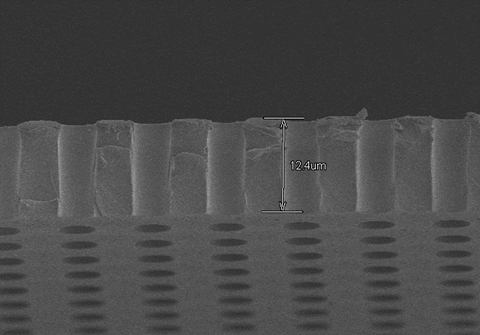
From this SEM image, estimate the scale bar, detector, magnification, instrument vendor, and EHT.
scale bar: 20000 nm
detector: SE(M)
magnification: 2 K X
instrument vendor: Hitachi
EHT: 3 kV